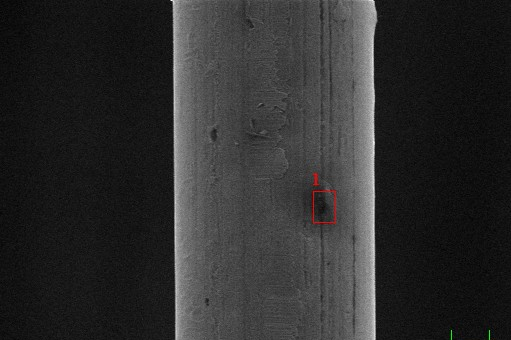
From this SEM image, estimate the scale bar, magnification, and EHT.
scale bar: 10000 nm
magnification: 1 K X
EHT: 20 kV